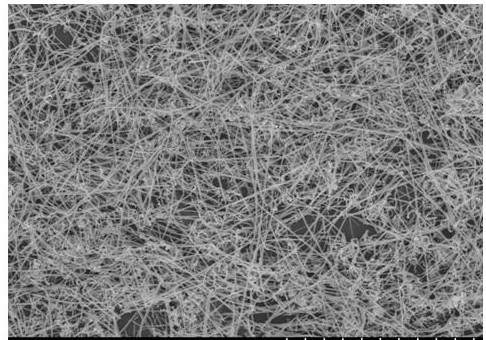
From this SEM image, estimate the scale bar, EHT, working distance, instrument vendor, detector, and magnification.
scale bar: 10000 nm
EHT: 15 kV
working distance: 8.9 mm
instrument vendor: Hitachi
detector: SE(U)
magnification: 5 K X